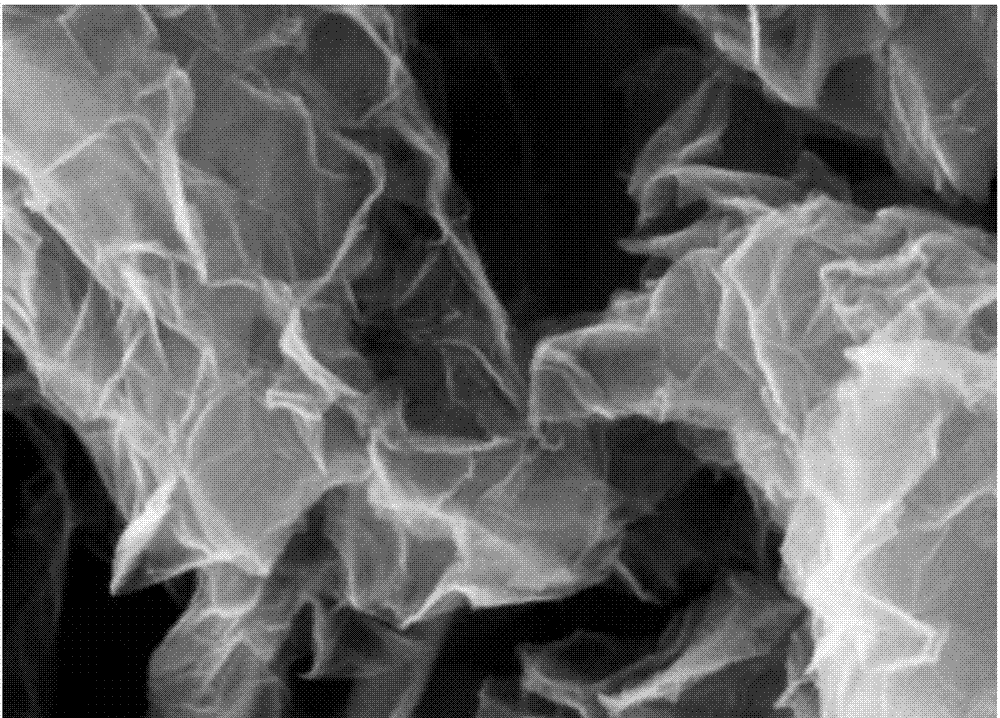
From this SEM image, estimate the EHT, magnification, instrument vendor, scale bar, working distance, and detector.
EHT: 5 kV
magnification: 100 K X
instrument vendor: Zeiss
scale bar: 100 nm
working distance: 3.8 mm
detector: InLens